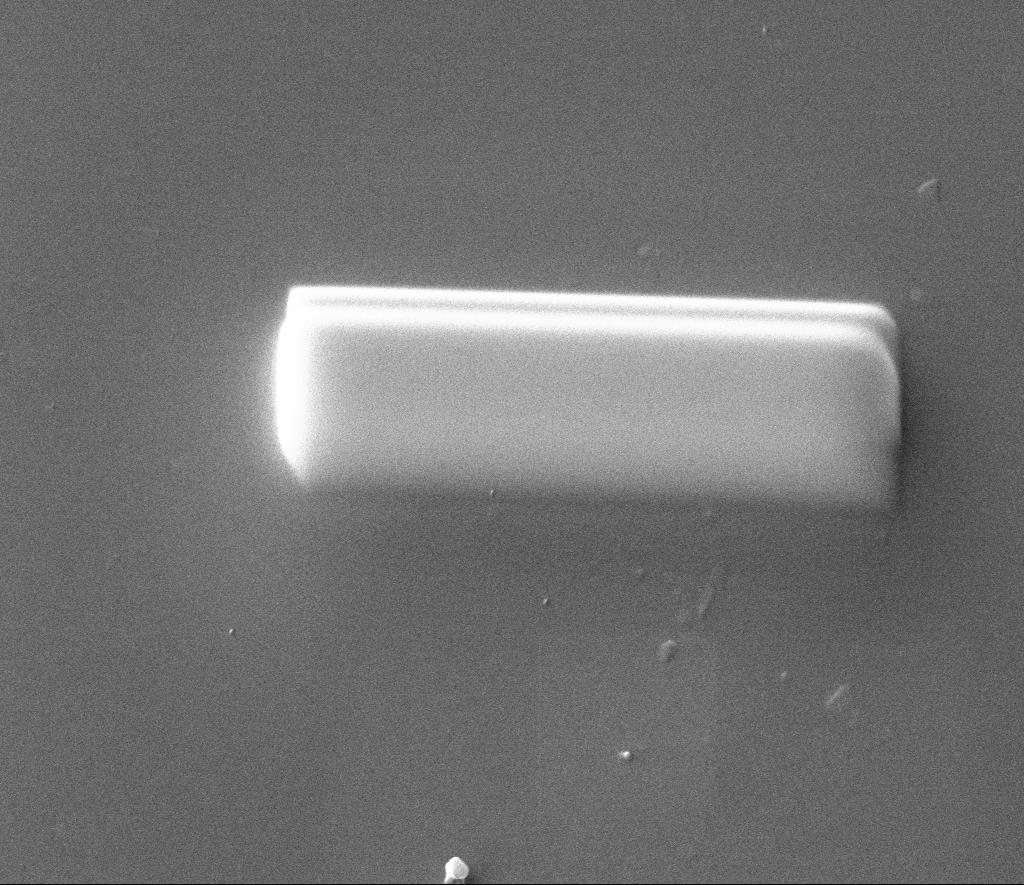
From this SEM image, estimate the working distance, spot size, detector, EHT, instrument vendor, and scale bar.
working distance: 10 mm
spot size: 4.5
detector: CDEM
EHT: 20 kV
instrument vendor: FEI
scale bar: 5000 nm